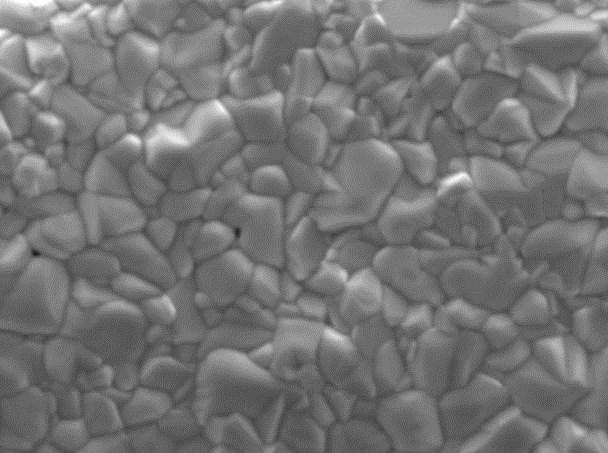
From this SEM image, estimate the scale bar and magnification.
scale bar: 1000 nm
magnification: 20 K X